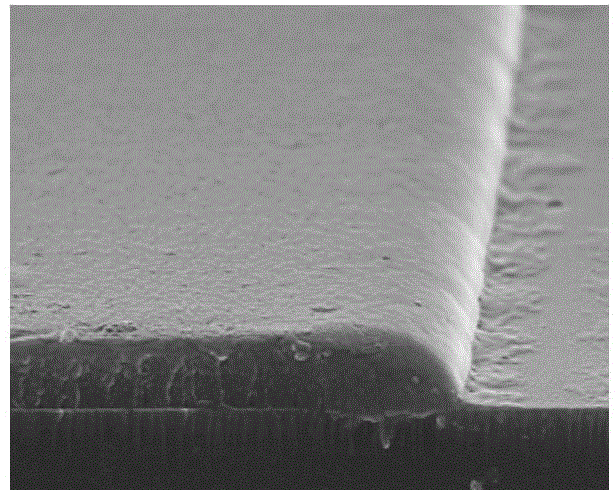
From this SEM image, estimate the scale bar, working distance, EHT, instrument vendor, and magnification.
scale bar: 10000 nm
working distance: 12.5 mm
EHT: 15 kV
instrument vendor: Hitachi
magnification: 5 K X